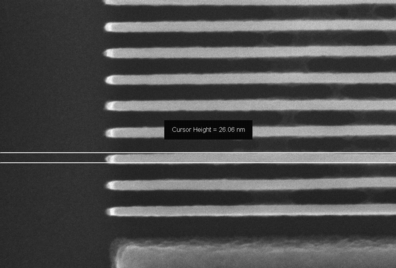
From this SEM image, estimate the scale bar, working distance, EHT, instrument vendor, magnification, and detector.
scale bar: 200 nm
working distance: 5 mm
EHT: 5 kV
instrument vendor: Zeiss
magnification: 366.37 K X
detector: InLens